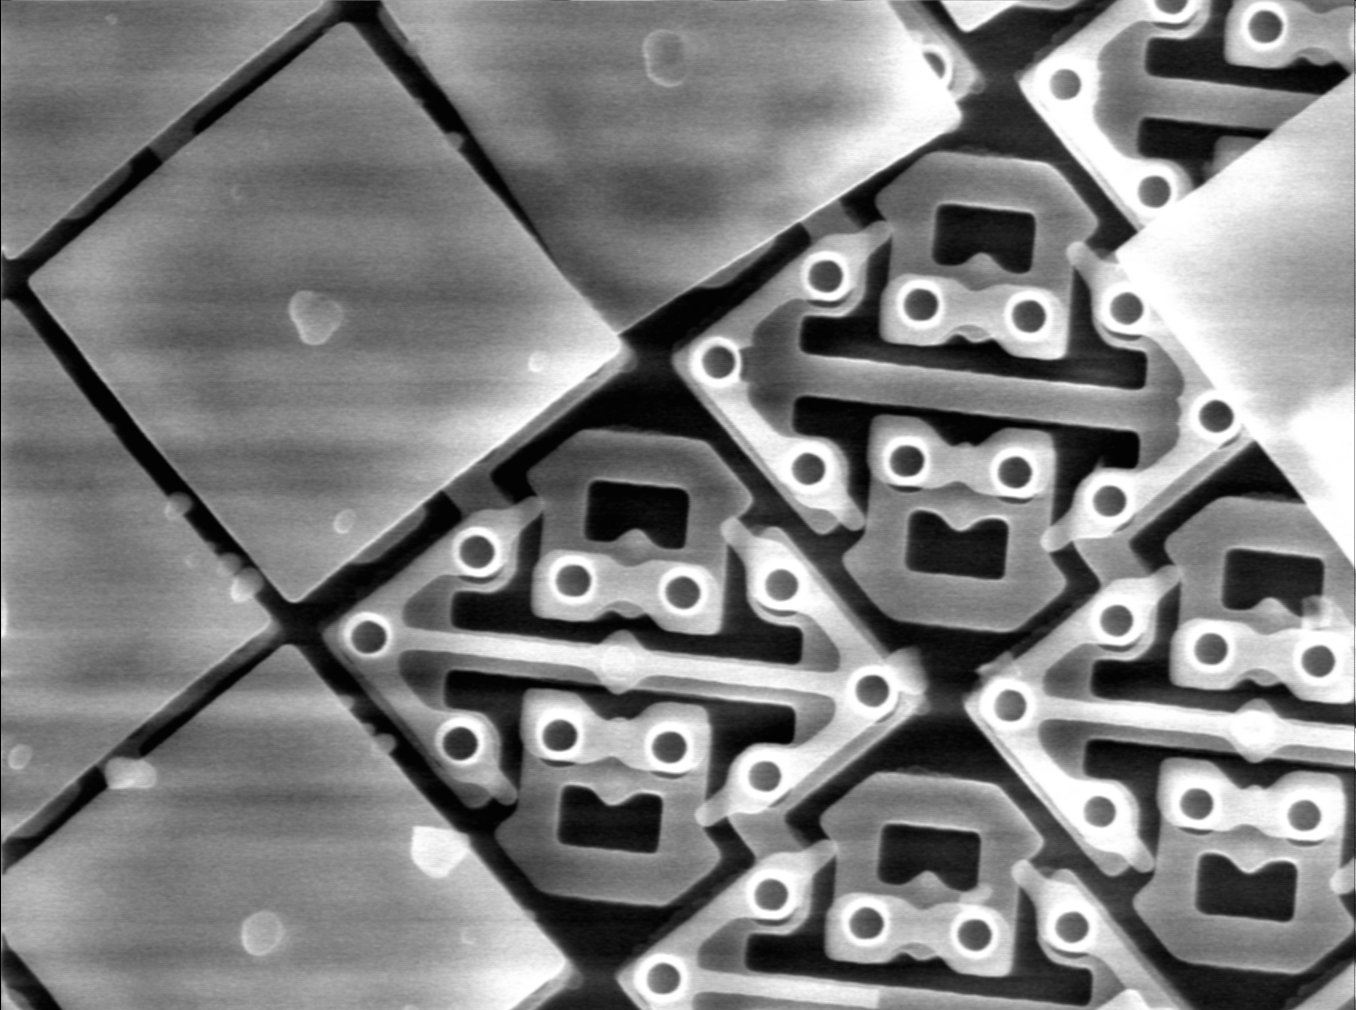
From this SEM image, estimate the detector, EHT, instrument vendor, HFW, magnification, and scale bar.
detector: SE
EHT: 15 kV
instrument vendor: other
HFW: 40 µm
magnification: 3 K X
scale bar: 3330 nm